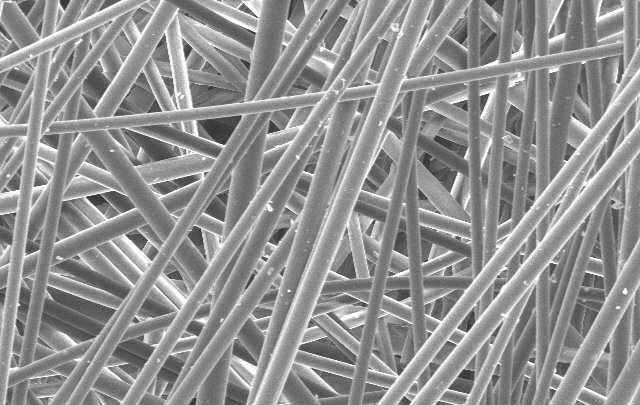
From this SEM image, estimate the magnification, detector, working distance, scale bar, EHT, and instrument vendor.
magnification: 0.5 K X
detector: SE(M)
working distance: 11.2 mm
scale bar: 100000 nm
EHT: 10 kV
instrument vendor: Hitachi